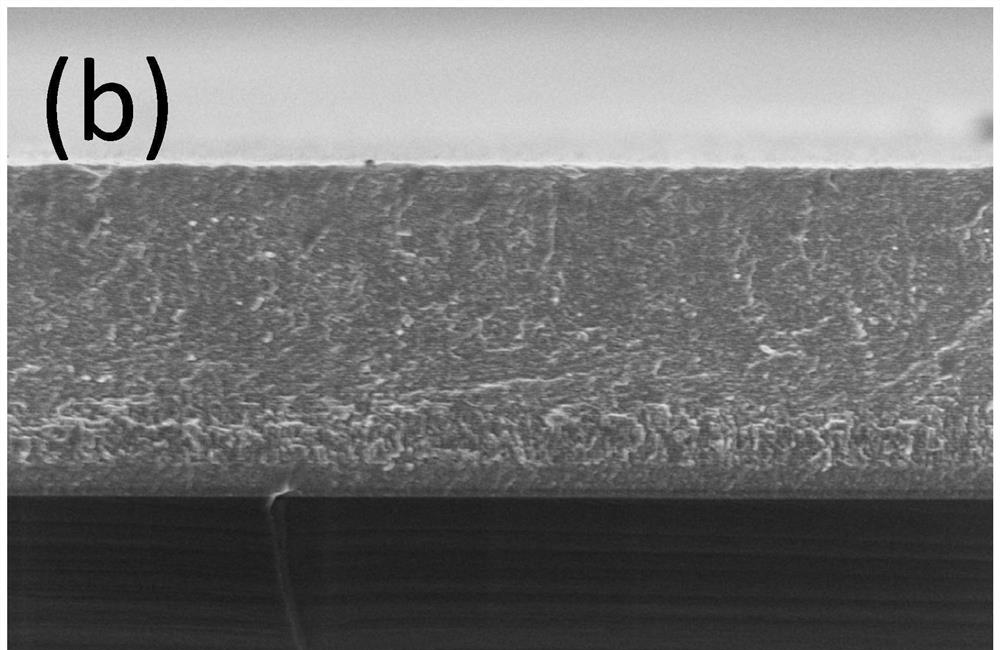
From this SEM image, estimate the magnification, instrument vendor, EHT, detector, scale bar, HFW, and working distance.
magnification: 20.001 K X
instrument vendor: Thermo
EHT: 2 kV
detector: TLD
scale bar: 1000 nm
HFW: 6.35 µm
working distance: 3.7 mm